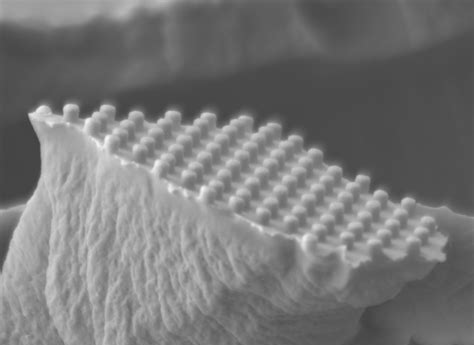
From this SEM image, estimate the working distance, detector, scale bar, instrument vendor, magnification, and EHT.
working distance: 6.7 mm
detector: LEI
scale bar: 1000 nm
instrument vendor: JEOL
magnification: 20 K X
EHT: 5 kV